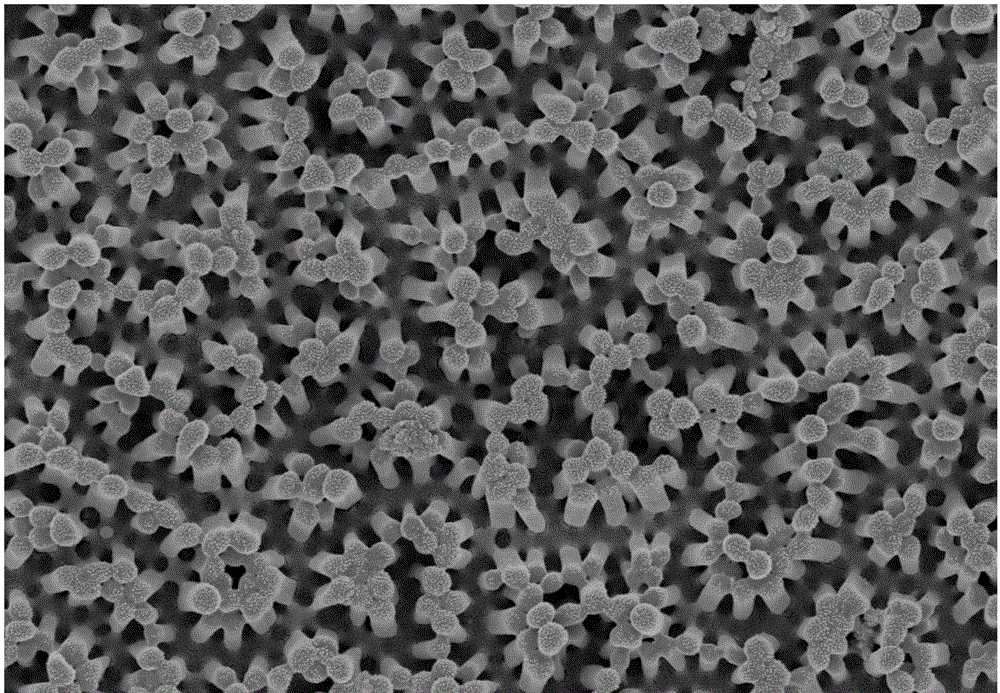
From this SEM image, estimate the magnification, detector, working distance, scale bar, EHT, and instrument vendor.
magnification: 10 K X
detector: InLens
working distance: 5.2 mm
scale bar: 1000 nm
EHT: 5 kV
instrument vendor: Zeiss